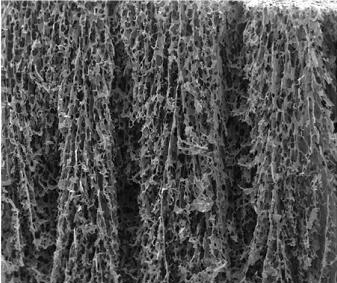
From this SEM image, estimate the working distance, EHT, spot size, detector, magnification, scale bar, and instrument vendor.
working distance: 13.1 mm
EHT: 10 kV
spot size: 3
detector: ETD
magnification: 0.15 K X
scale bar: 500000 nm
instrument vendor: FEI